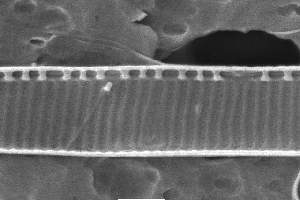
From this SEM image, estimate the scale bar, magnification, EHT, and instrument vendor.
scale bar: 1000 nm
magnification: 20 K X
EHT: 10 kV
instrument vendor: JEOL